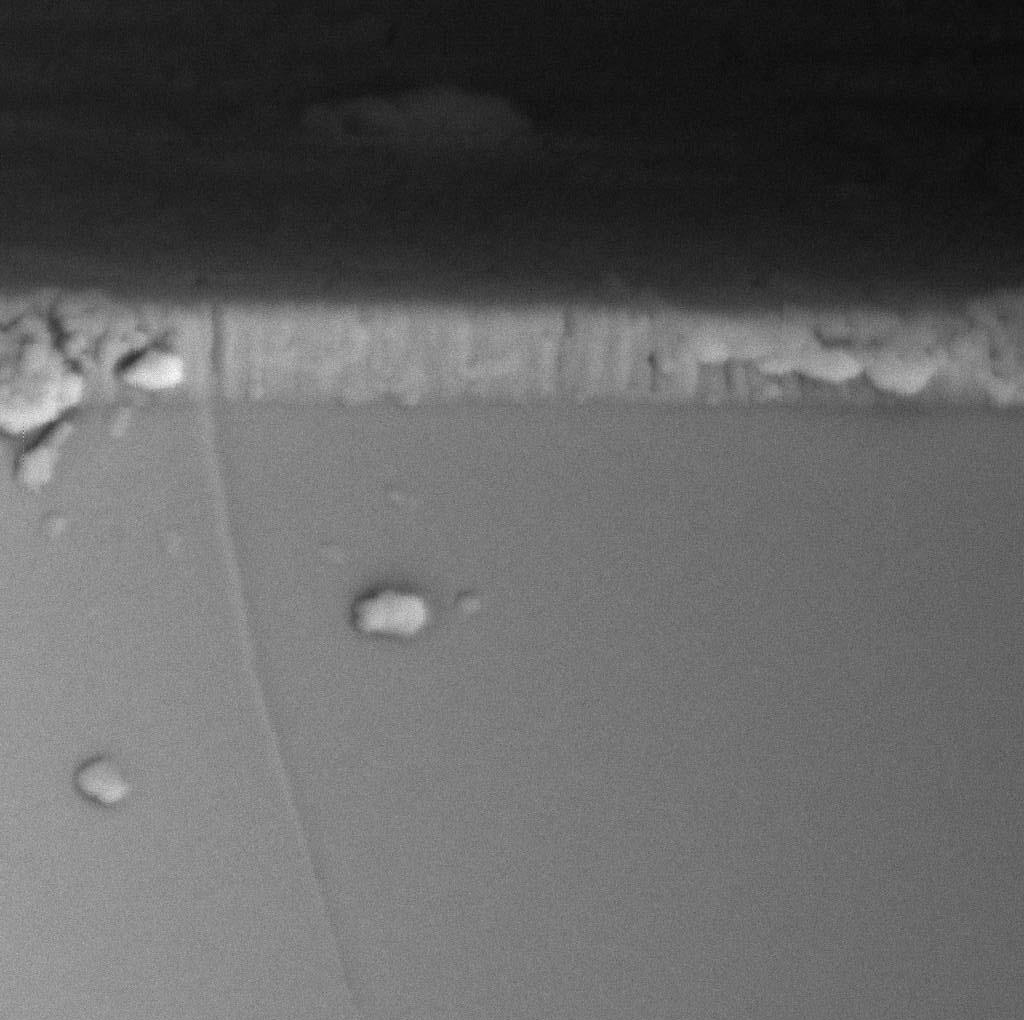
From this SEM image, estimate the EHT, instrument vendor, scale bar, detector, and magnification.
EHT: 30 kV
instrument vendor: Tescan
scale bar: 2000 nm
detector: BE Detector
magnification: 60.02 K X